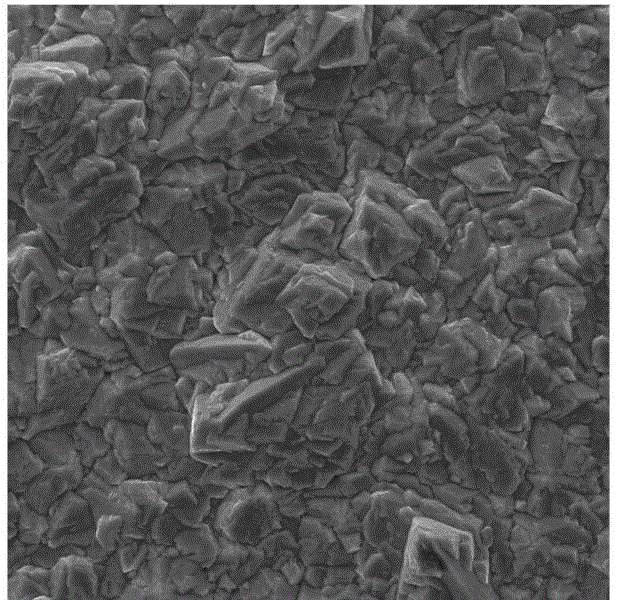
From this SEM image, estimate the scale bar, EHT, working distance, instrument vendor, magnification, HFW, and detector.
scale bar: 20000 nm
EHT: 30 kV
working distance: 7.98 mm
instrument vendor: Tescan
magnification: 2 K X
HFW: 104 µm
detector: SE